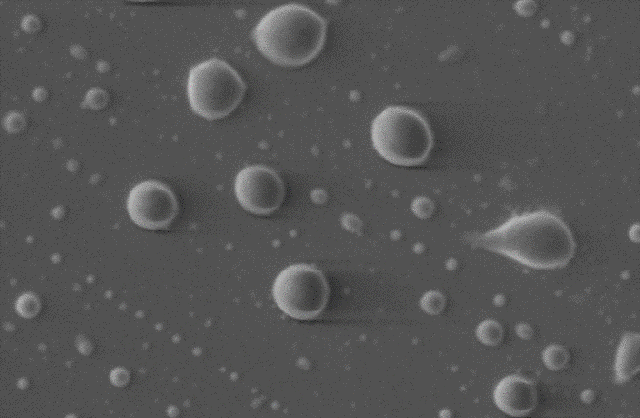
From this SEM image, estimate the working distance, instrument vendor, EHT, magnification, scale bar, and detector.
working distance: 12 mm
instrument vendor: Zeiss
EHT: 5 kV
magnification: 14 K X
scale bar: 2000 nm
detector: SE1